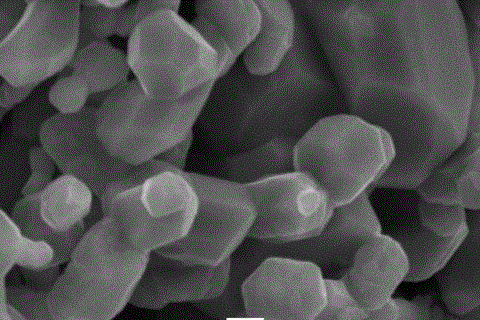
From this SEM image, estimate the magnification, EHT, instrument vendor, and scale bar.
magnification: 10 K X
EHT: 15 kV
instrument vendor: other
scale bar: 1000 nm